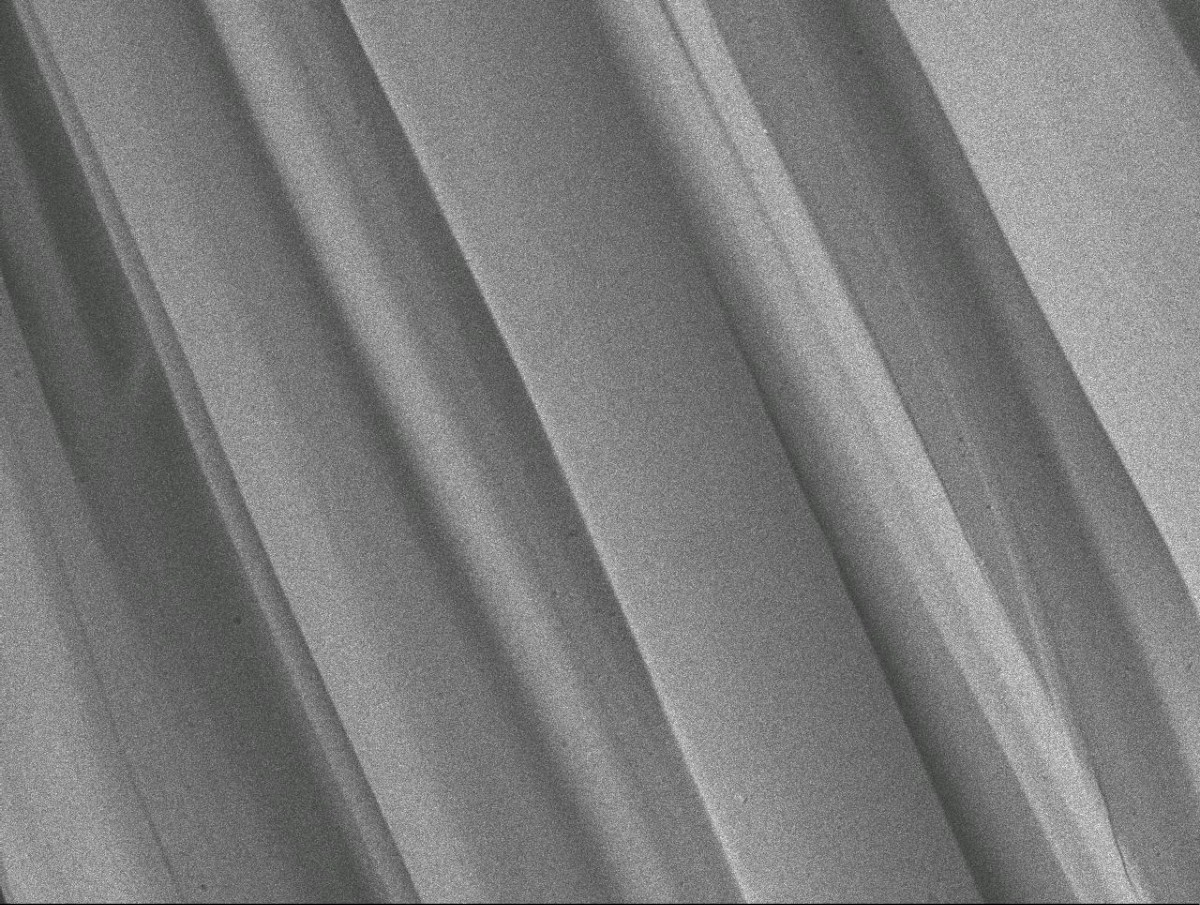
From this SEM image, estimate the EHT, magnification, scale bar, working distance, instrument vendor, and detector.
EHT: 5 kV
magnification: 2 K X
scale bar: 10000 nm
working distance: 9.3 mm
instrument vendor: JEOL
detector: LEI